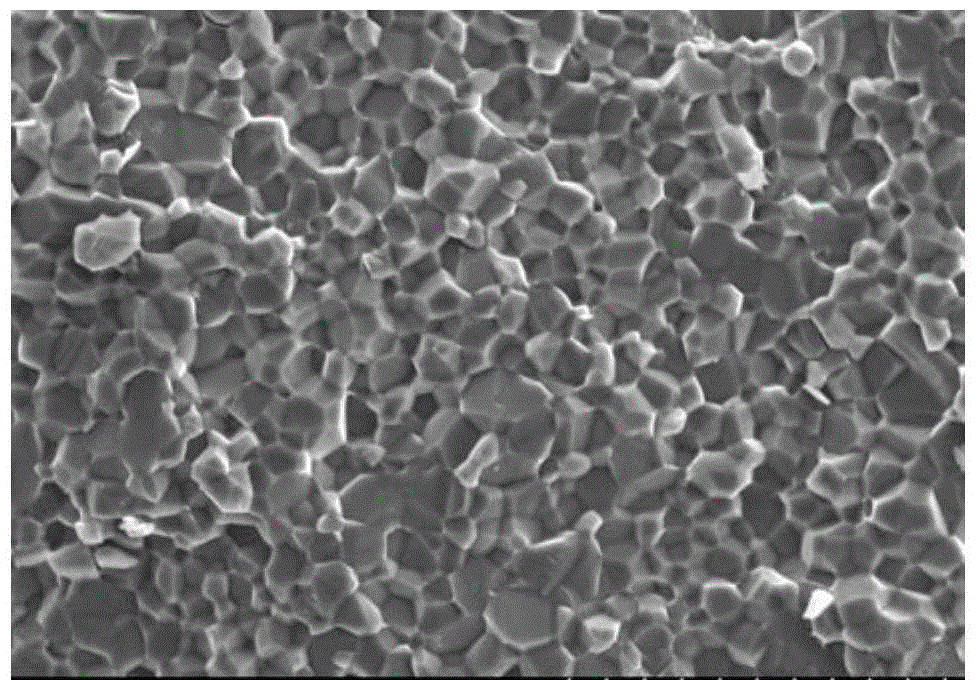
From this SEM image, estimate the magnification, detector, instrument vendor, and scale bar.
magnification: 1 K X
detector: SE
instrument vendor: Hitachi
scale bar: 50000 nm